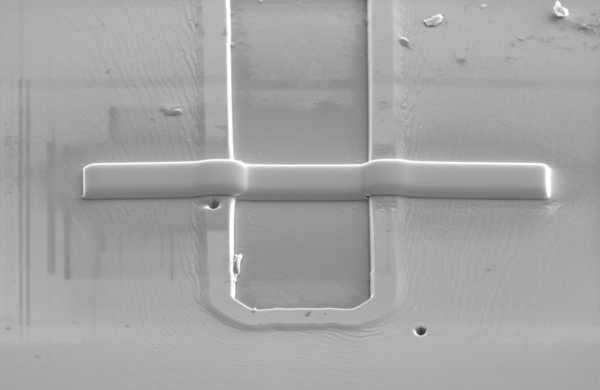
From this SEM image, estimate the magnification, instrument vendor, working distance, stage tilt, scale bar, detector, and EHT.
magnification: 8 K X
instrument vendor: FEI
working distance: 7.1 mm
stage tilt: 52°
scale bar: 5000 nm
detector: ETD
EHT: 5 kV